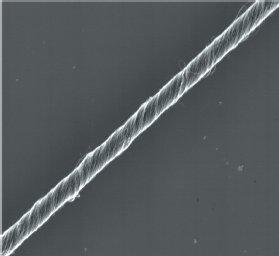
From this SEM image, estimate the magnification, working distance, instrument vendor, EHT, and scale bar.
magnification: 2.3 K X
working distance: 17 mm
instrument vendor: Hitachi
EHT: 2 kV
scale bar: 1000 nm